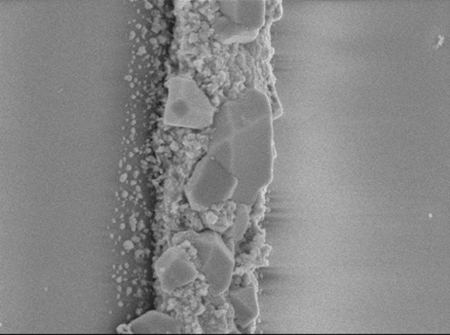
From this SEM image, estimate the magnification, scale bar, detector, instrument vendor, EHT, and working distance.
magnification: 15 K X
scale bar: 1000 nm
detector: SEI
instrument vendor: JEOL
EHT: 5 kV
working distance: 14 mm